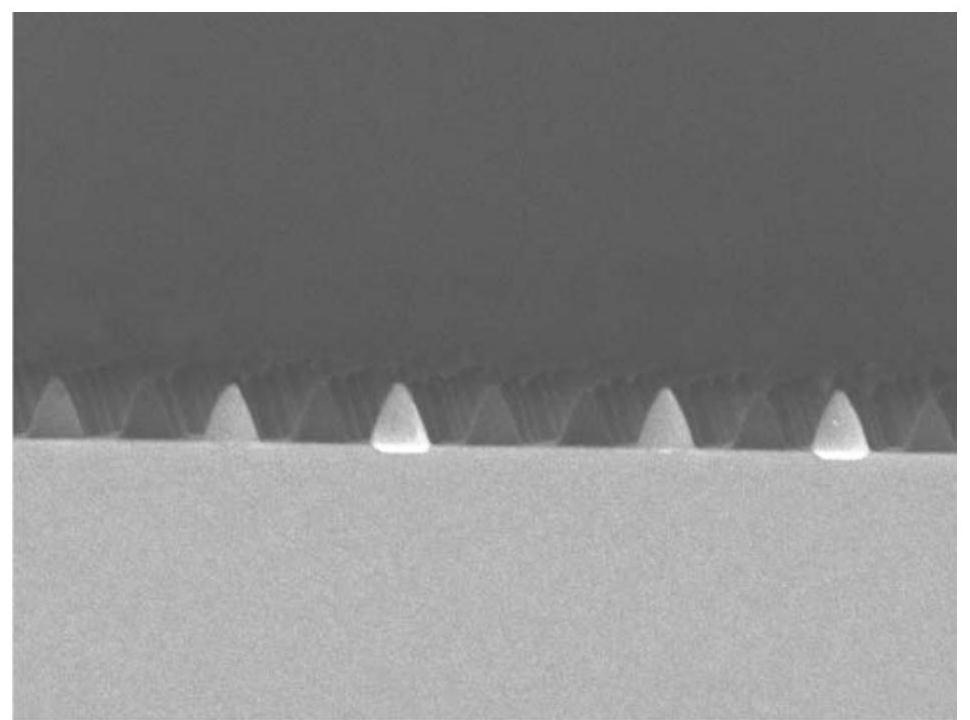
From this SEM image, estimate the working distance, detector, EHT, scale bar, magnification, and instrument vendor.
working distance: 7.1 mm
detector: SEI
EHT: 3 kV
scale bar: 1000 nm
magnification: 25 K X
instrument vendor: JEOL